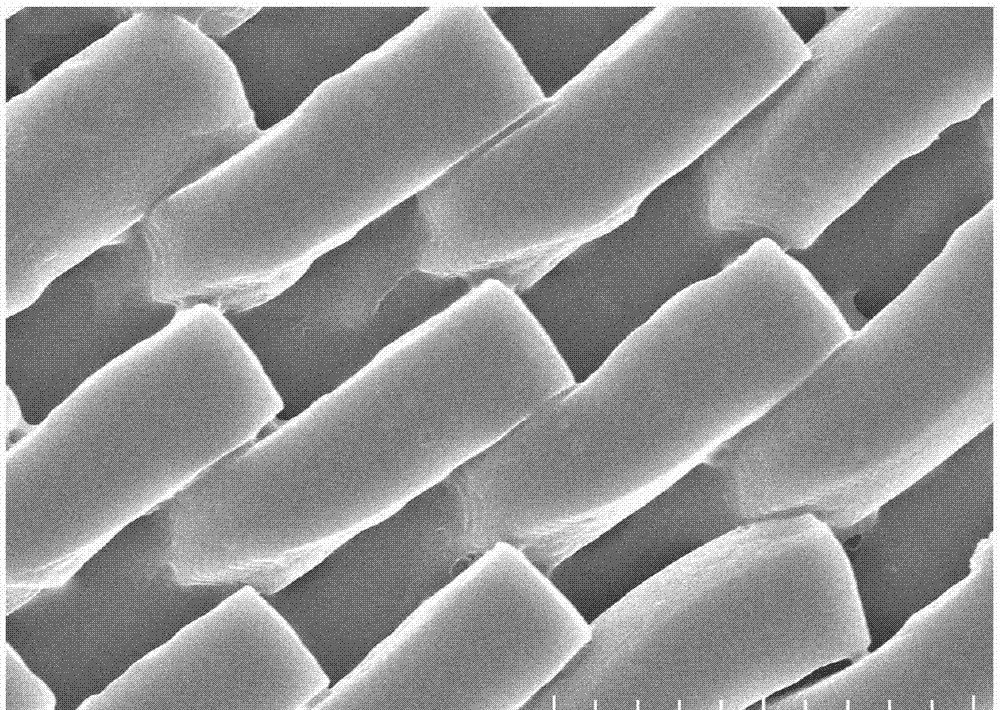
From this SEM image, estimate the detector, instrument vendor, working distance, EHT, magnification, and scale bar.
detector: SE(U)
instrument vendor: Hitachi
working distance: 8.9 mm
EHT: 15 kV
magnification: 1.8 K X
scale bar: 30000 nm